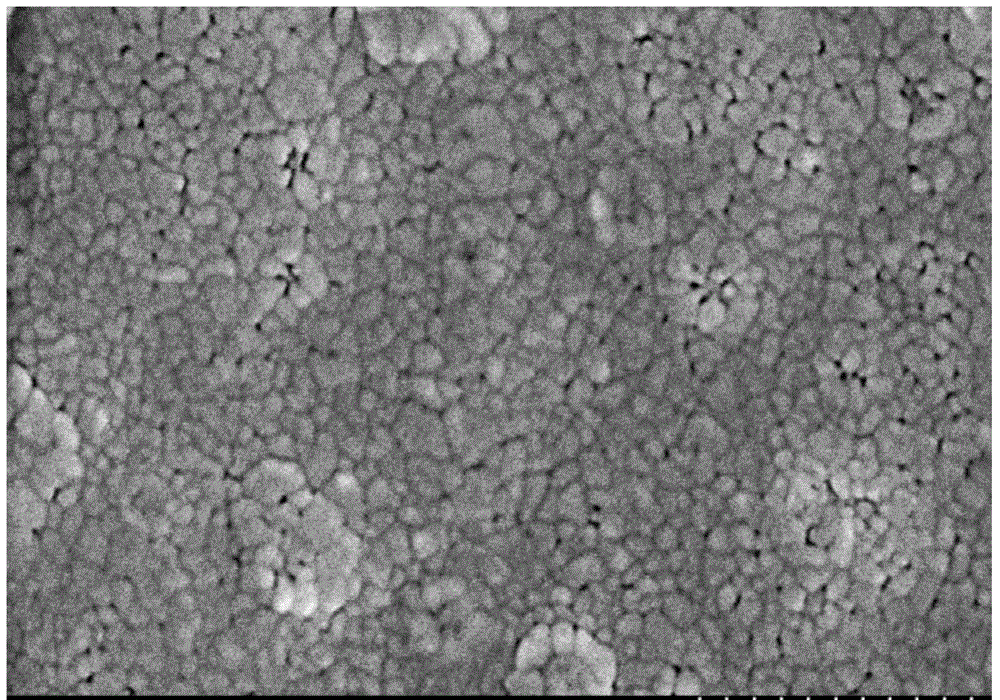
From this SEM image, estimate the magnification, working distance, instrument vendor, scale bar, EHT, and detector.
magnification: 70 K X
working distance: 10.4 mm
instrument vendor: Hitachi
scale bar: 500 nm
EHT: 3 kV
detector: SE(M)